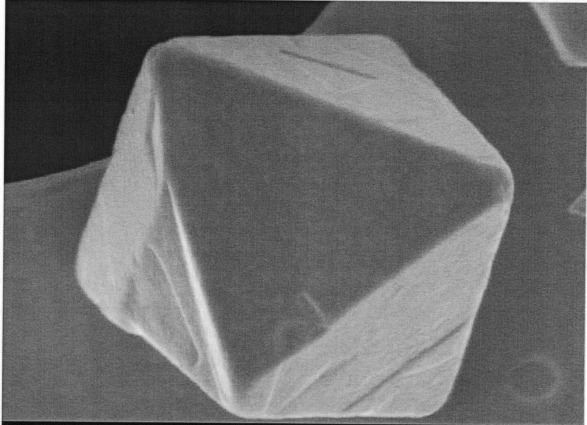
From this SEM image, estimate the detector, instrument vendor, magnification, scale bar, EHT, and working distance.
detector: SEI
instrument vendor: JEOL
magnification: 50 K X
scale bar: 100 nm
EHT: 1 kV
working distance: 3 mm